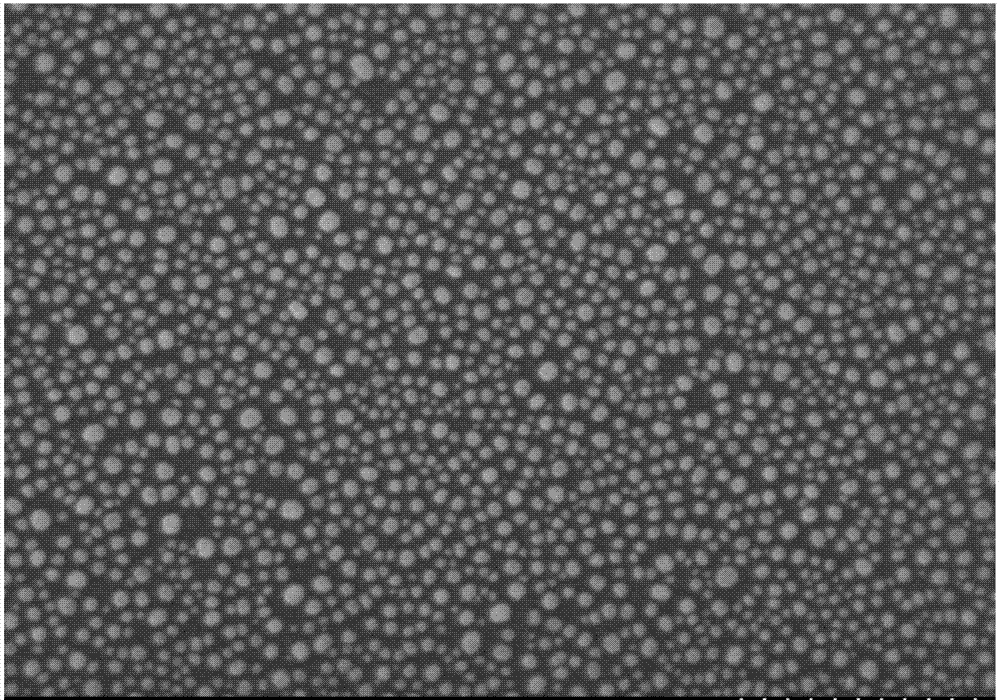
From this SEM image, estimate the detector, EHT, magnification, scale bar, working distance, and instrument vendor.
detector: SE(U)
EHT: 7 kV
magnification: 60 K X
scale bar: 500 nm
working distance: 9 mm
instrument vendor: Hitachi